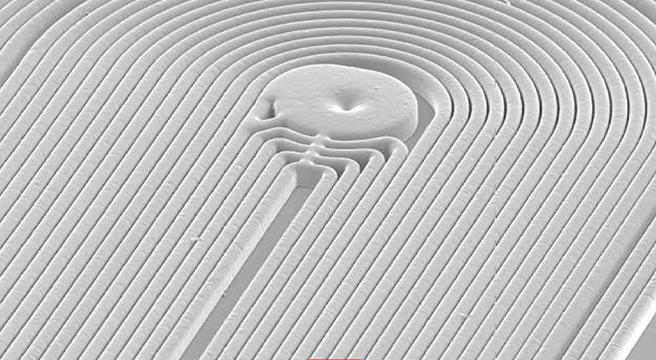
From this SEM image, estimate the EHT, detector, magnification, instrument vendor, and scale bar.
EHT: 15 kV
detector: SEI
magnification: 0.1 K X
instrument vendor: JEOL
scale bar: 100000 nm